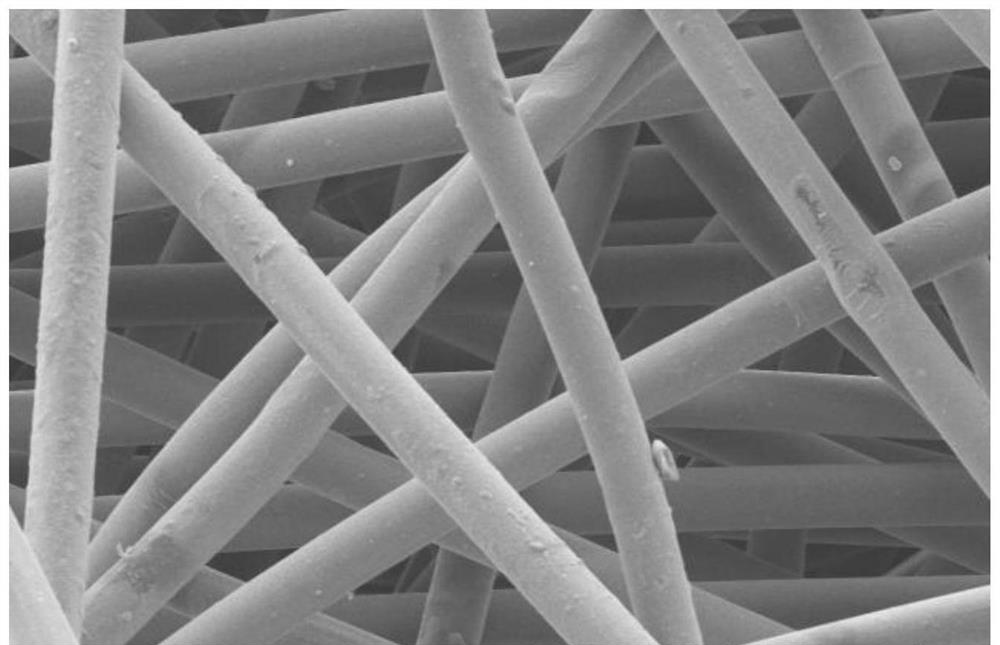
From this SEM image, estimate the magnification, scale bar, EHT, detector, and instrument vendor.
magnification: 0.5 K X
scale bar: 100000 nm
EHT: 10 kV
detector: SE(M)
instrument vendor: Hitachi